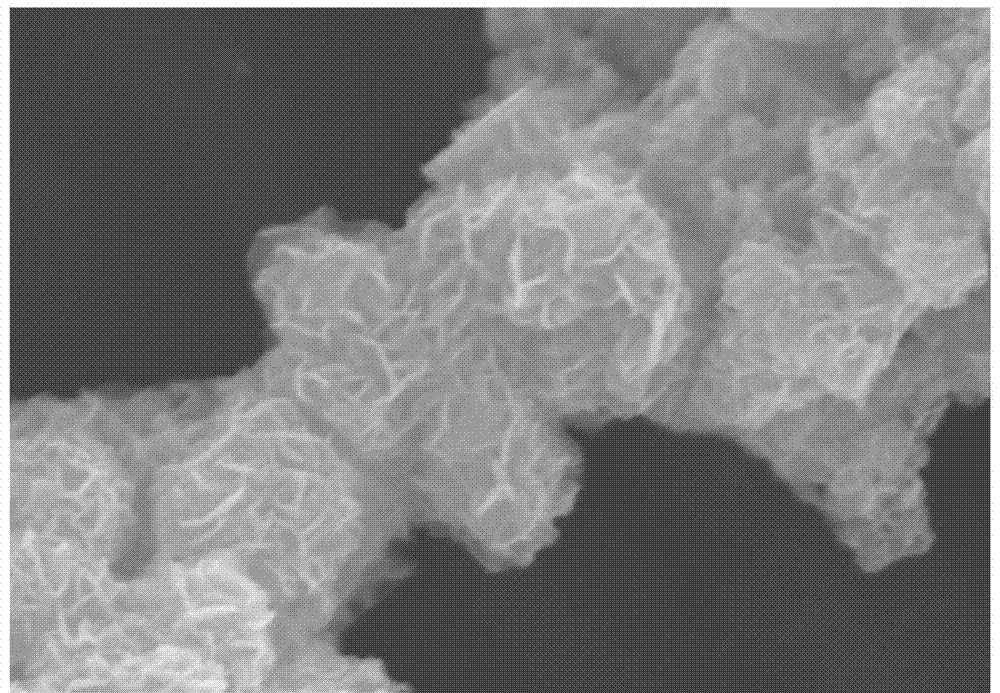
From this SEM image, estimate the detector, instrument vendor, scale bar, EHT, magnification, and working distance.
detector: SE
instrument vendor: Hitachi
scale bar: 2000 nm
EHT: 15 kV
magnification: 20 K X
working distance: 10.2 mm